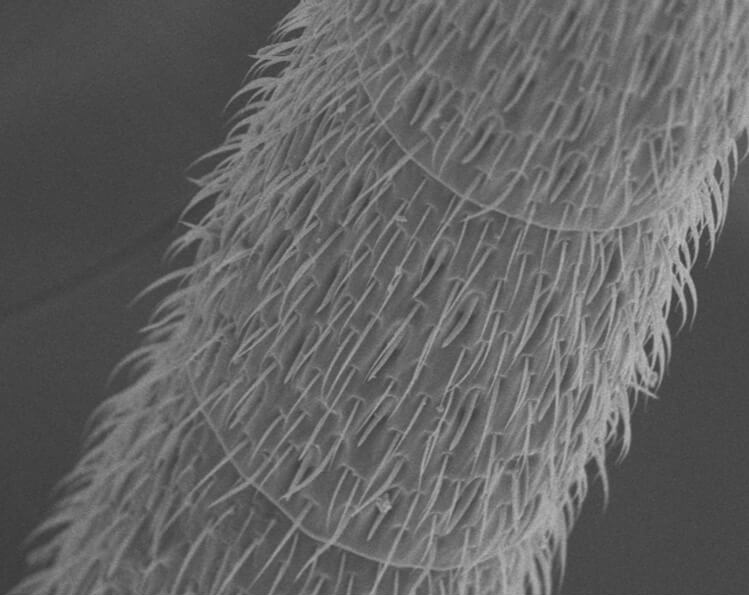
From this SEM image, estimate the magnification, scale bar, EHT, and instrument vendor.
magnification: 0.6 K X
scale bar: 50000 nm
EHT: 10 kV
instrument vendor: JEOL